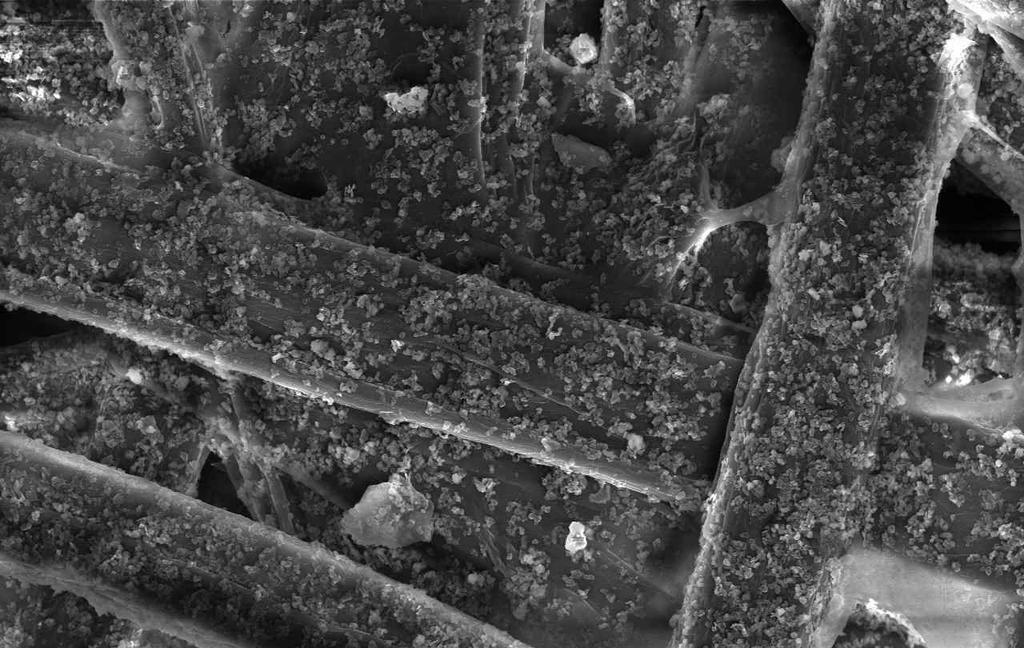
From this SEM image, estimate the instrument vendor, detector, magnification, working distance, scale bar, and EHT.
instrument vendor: Hitachi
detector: SE(U)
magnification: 1 K X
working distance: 11.8 mm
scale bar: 50000 nm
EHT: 15 kV